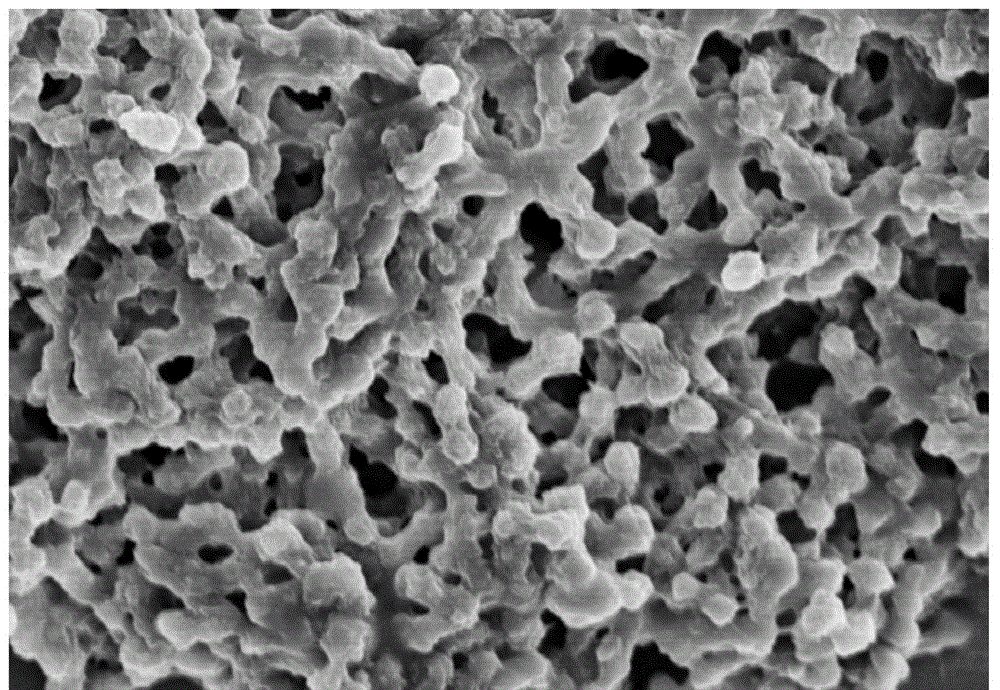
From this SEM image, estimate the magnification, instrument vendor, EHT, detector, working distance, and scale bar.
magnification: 10 K X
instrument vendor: Hitachi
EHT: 5 kV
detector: SE(M)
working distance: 7.8 mm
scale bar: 5000 nm